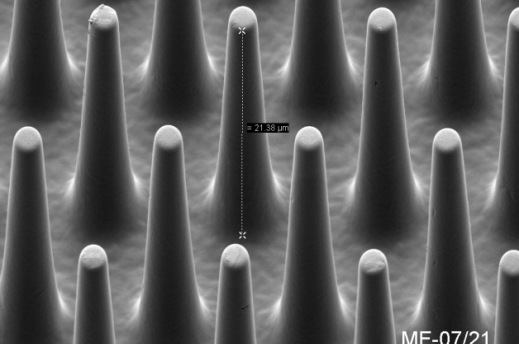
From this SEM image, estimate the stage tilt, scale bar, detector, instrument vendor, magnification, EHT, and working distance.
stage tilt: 45°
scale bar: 2000 nm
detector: SE2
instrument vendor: Zeiss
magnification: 5 K X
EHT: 2 kV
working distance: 7.2 mm